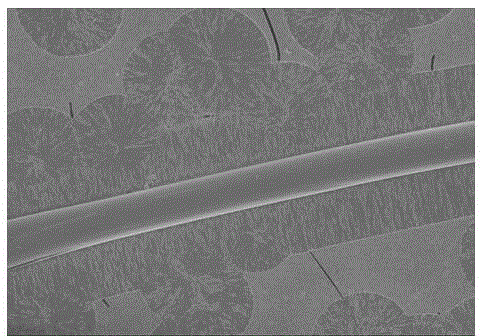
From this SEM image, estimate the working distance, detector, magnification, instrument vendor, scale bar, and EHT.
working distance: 9.6 mm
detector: SE(M)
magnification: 0.5 K X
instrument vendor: Hitachi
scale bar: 100000 nm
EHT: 3 kV